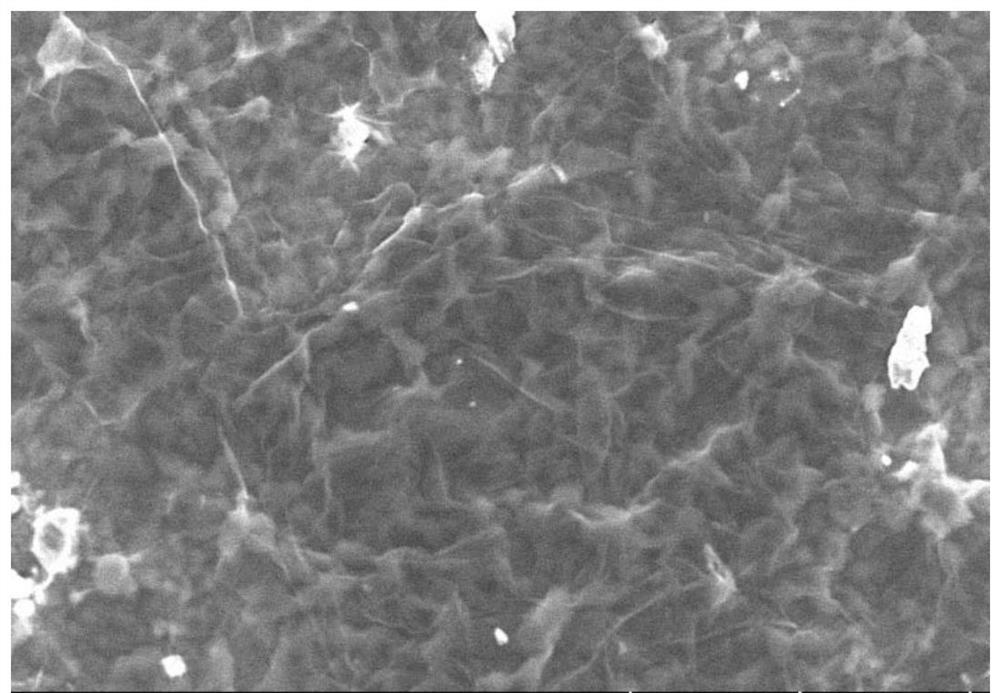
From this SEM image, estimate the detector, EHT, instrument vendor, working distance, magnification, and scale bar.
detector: SE(UL)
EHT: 10 kV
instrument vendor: Hitachi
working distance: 12.8 mm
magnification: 2.2 K X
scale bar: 20000 nm